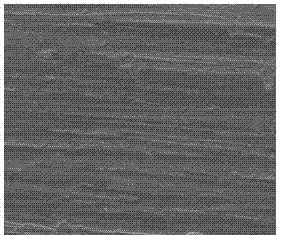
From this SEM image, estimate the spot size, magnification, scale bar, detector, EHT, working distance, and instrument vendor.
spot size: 3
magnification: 1 K X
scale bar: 50000 nm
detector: ETD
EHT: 10 kV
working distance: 7.7 mm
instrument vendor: FEI